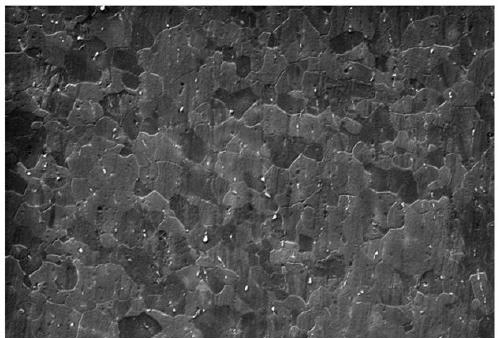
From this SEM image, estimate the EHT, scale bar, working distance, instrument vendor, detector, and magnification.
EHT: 20 kV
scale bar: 10000 nm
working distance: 12.5 mm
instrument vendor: Zeiss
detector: SE1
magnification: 1 K X